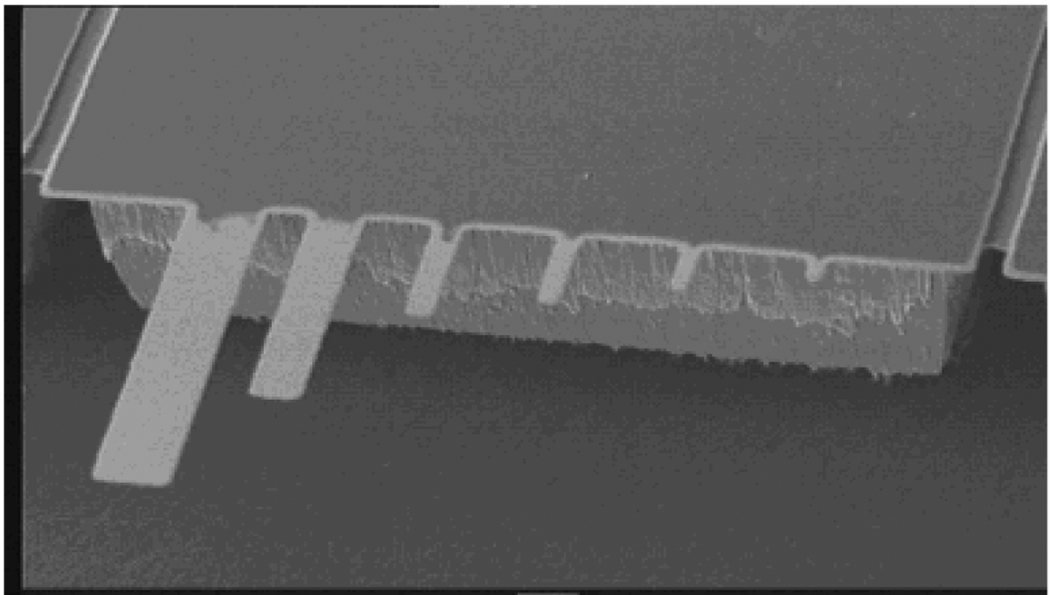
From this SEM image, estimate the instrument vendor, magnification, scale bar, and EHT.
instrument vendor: other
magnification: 0.072 K X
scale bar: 100000 nm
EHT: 25 kV